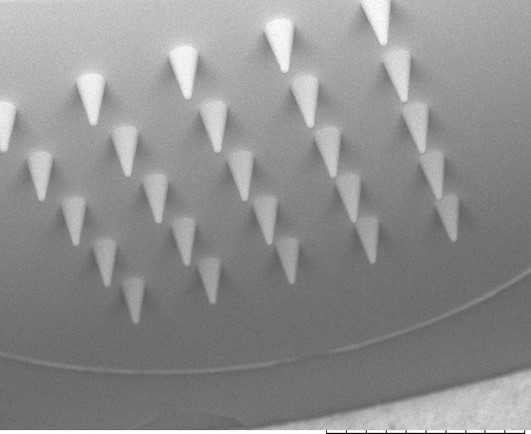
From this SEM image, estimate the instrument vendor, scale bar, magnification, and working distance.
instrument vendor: Hitachi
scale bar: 2000000 nm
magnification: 0.03 K X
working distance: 10.3 mm